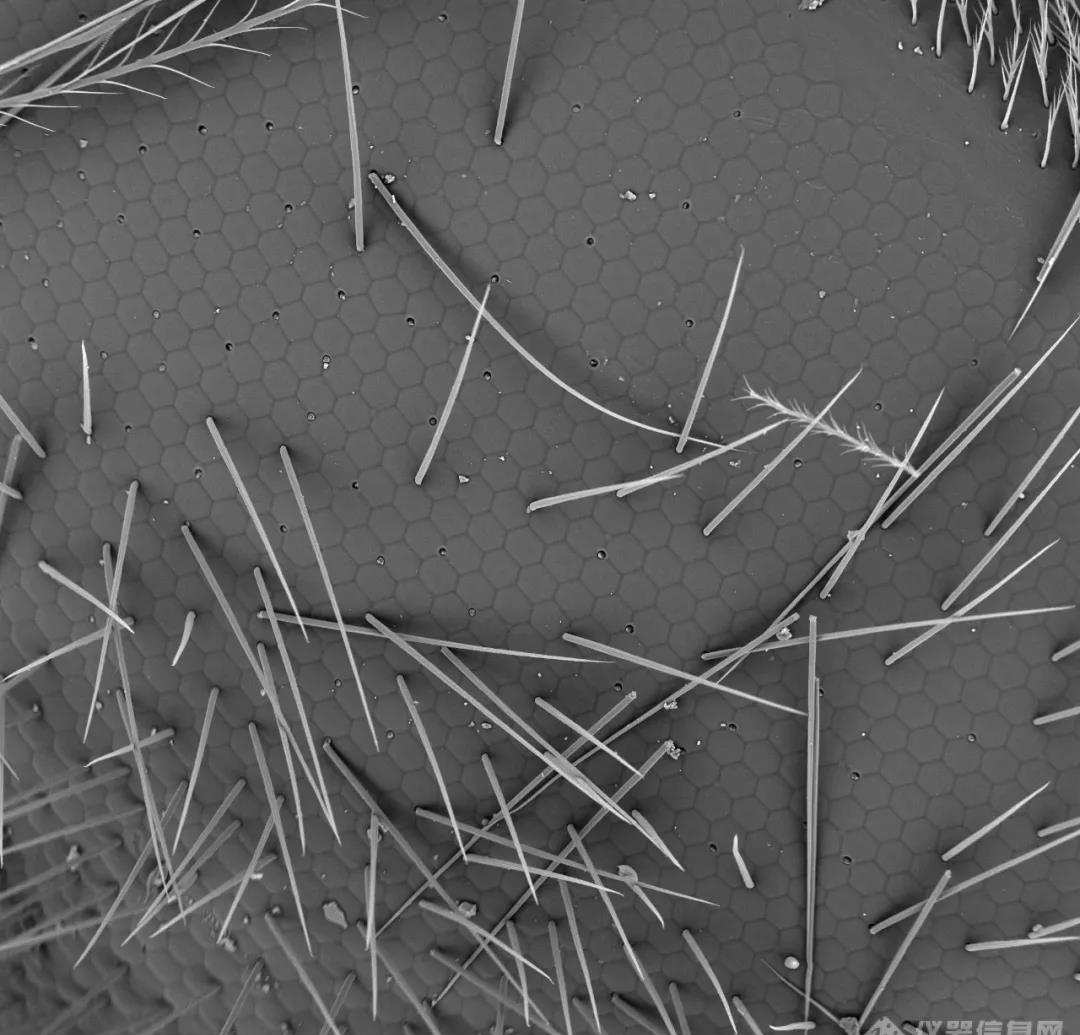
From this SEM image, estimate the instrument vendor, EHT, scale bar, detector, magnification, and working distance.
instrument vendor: other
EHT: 15 kV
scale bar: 100000 nm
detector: BSE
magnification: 0.5 K X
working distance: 5.7 mm